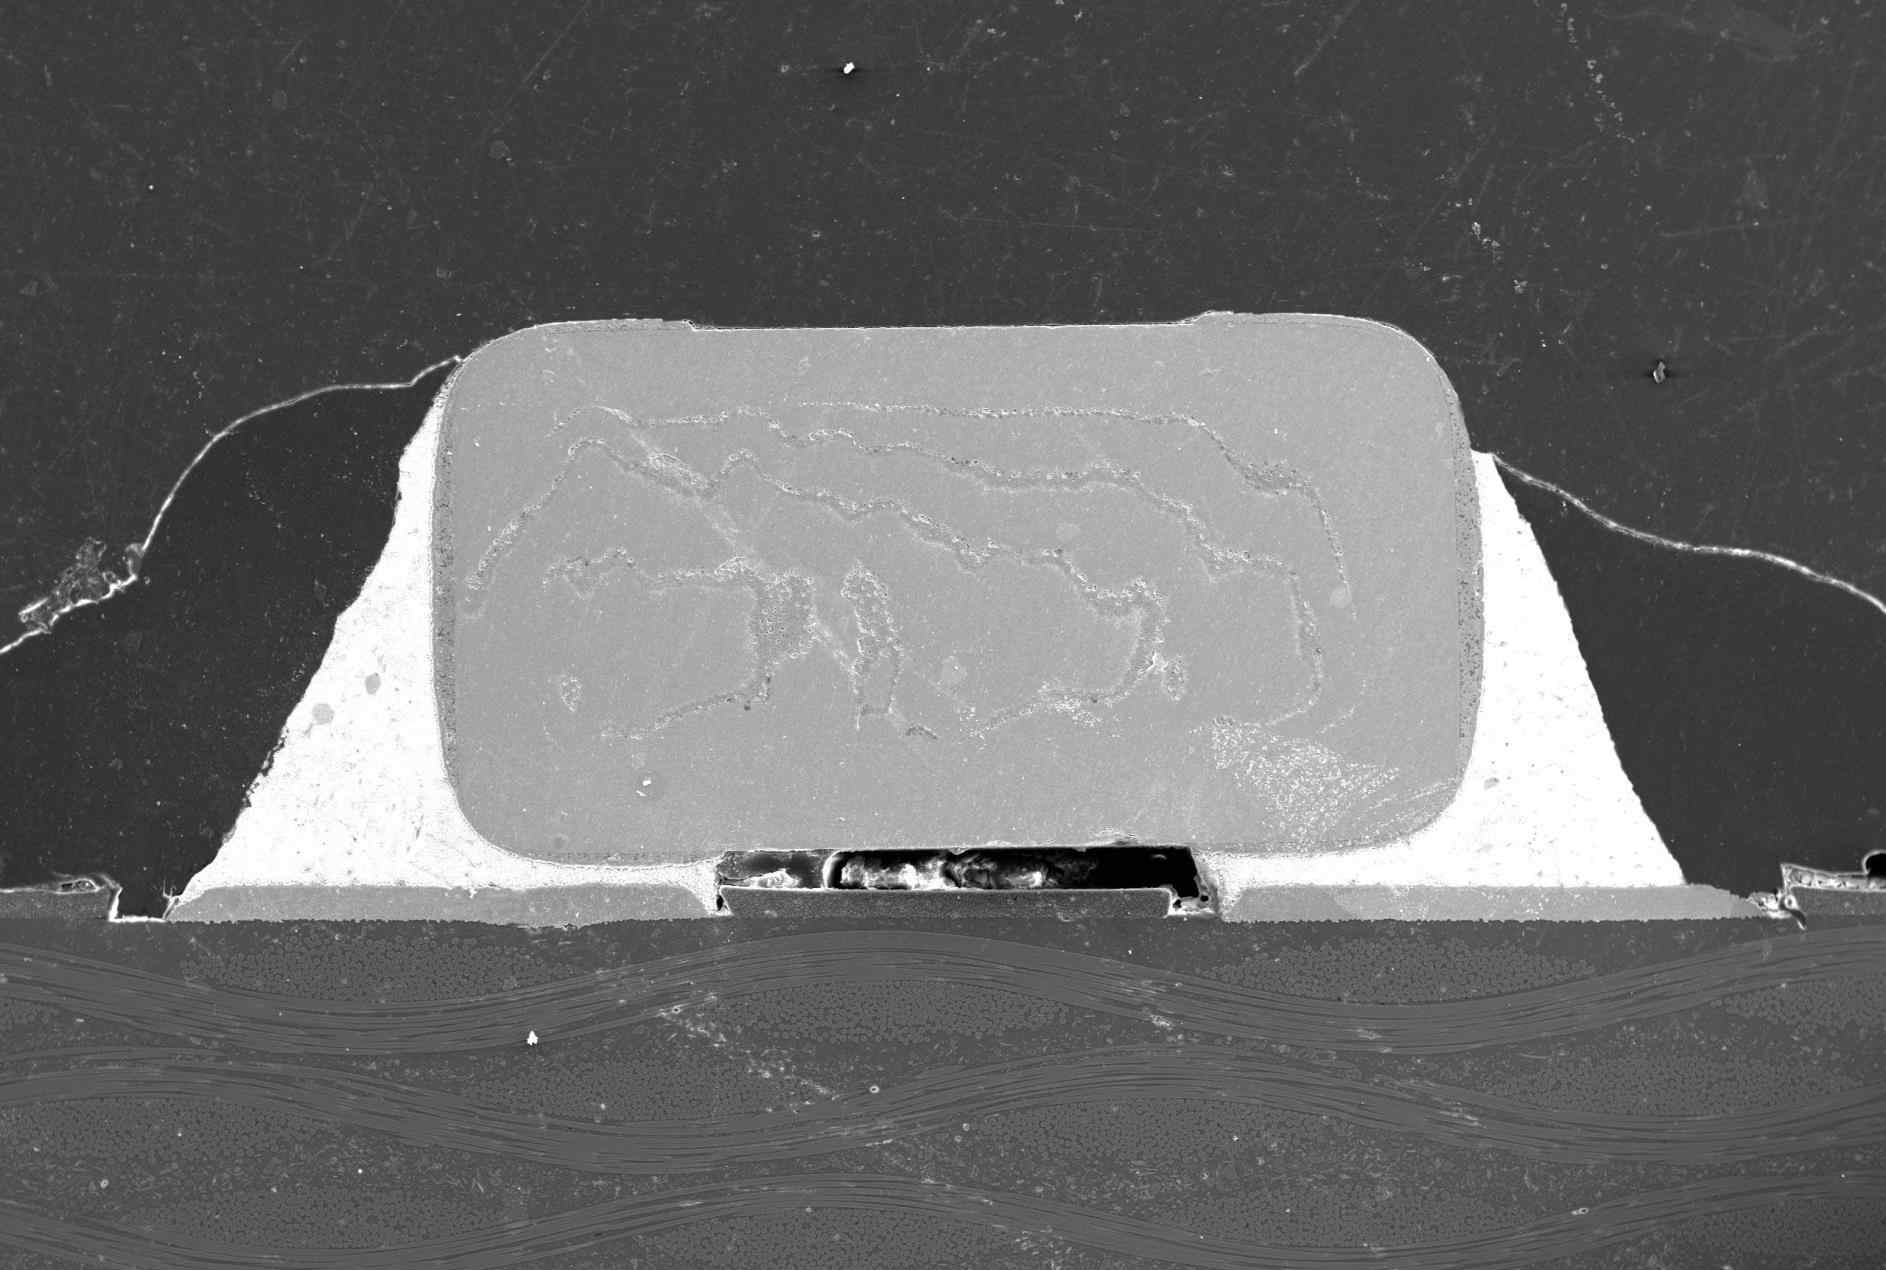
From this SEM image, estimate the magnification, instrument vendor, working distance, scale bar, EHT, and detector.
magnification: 0.045 K X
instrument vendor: JEOL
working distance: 10 mm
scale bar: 500000 nm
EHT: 20 kV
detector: SEI